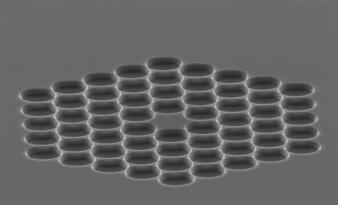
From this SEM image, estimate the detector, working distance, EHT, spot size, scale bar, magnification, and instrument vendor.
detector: SE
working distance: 14.8 mm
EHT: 15 kV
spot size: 4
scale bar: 10000 nm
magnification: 2.291 K X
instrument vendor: FEI